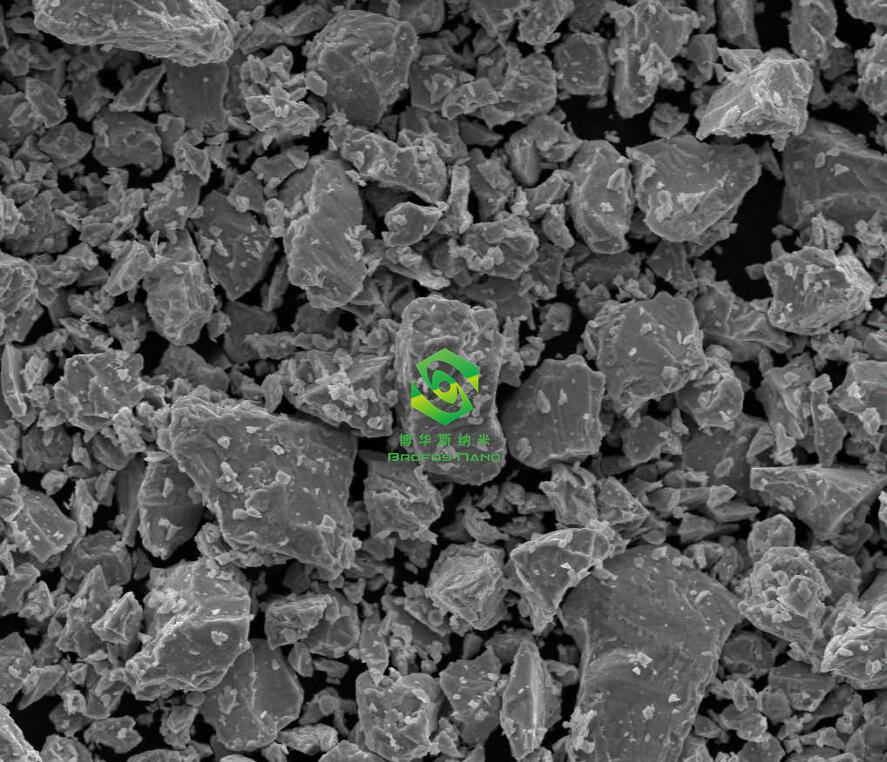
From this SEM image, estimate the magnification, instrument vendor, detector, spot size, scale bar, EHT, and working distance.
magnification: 2 K X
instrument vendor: FEI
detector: ETD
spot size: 4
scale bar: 50000 nm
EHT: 20 kV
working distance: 10.3 mm